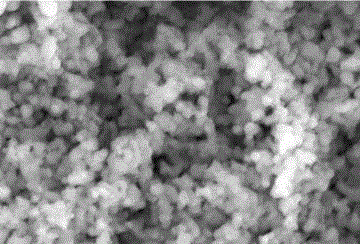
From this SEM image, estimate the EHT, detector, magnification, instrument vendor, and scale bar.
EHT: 20 kV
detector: SEI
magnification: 16 K X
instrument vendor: JEOL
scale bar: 1000 nm